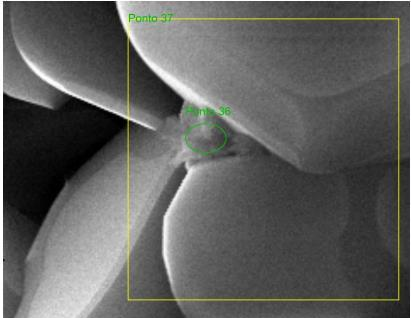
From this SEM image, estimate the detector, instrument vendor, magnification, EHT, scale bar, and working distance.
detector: SE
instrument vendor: other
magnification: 16.516 K X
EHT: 30 kV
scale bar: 1000 nm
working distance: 13.7 mm